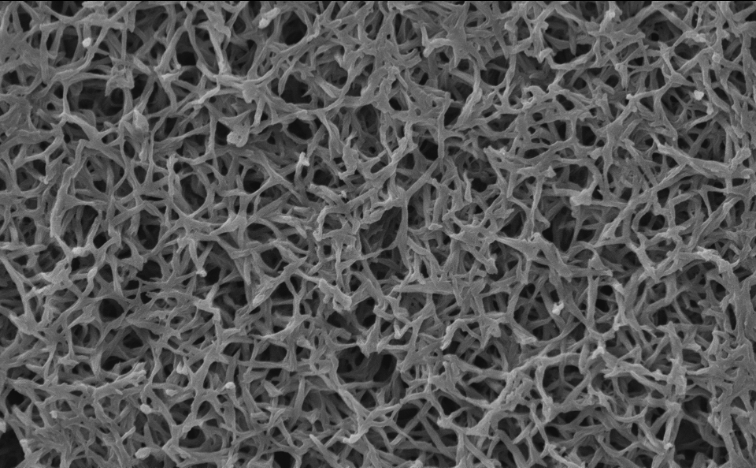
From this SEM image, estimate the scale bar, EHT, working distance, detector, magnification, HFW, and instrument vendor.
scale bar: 8000 nm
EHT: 10 kV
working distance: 4.894 mm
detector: SED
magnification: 5 K X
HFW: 25.4 µm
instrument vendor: Thermo